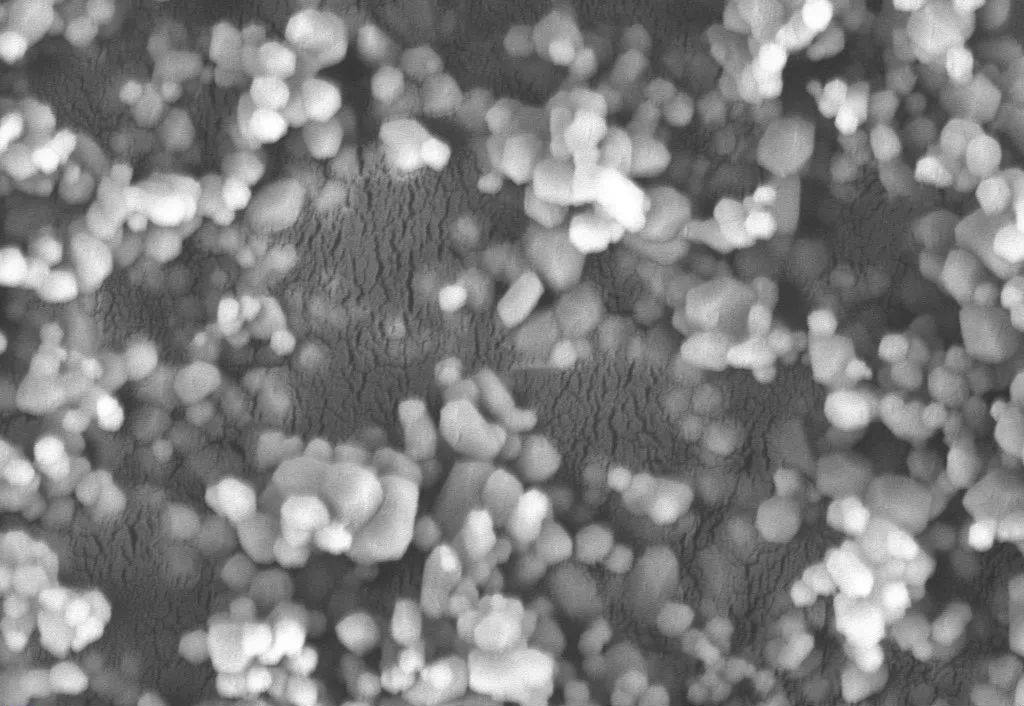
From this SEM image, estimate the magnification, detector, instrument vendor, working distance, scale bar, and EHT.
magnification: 20 K X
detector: SE2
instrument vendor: Zeiss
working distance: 5.5 mm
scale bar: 400 nm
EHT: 5 kV